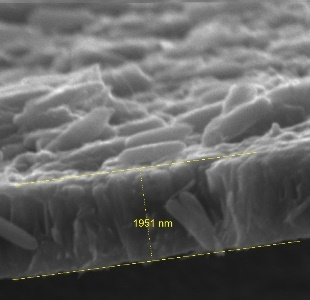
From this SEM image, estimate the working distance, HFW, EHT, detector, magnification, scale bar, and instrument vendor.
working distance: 5.88 mm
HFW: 6.46 µm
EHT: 15 kV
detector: SE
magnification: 32.2 K X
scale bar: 1000 nm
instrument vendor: Tescan